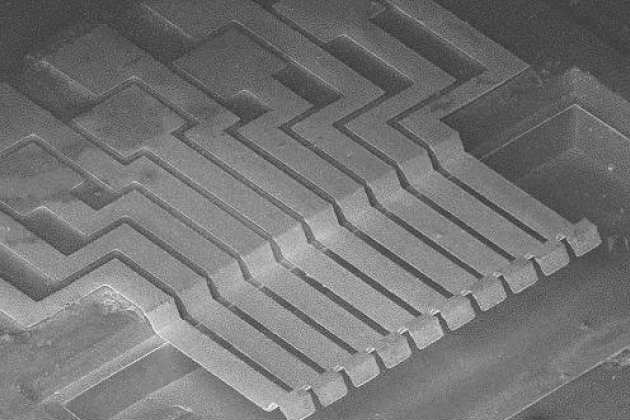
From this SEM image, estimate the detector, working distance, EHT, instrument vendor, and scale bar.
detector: SE1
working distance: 59 mm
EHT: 15 kV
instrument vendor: Zeiss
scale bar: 300000 nm